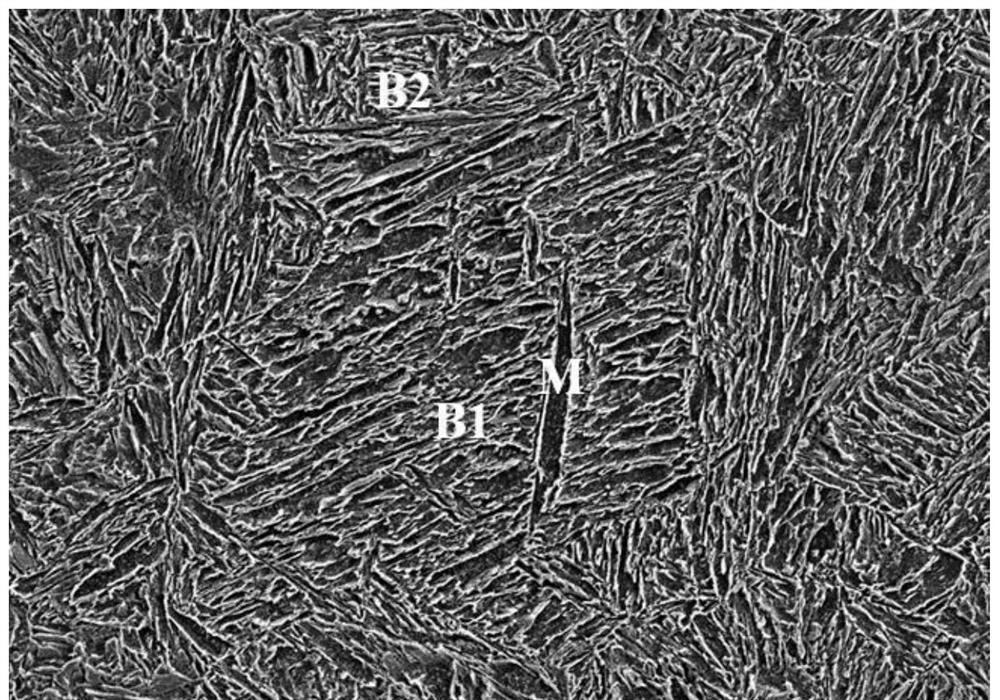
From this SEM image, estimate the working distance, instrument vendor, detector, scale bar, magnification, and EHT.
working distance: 8.2 mm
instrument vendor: Hitachi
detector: SE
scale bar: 20000 nm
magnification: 2 K X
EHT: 5 kV